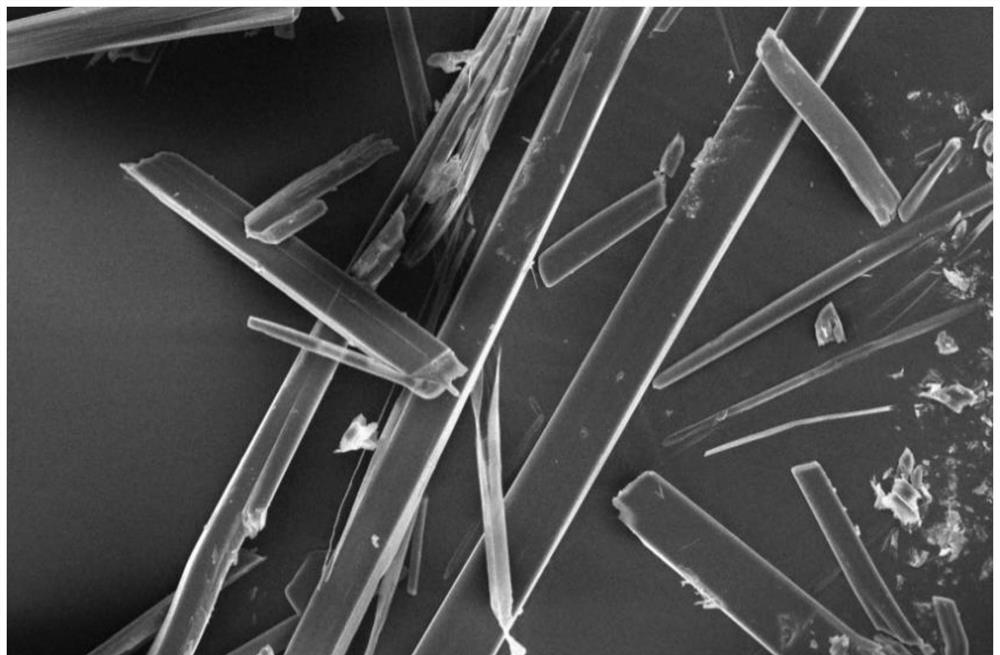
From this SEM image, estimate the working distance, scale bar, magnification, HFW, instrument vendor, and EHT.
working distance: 11.9 mm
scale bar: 300000 nm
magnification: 0.5 K X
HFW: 829 µm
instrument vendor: Thermo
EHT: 20 kV